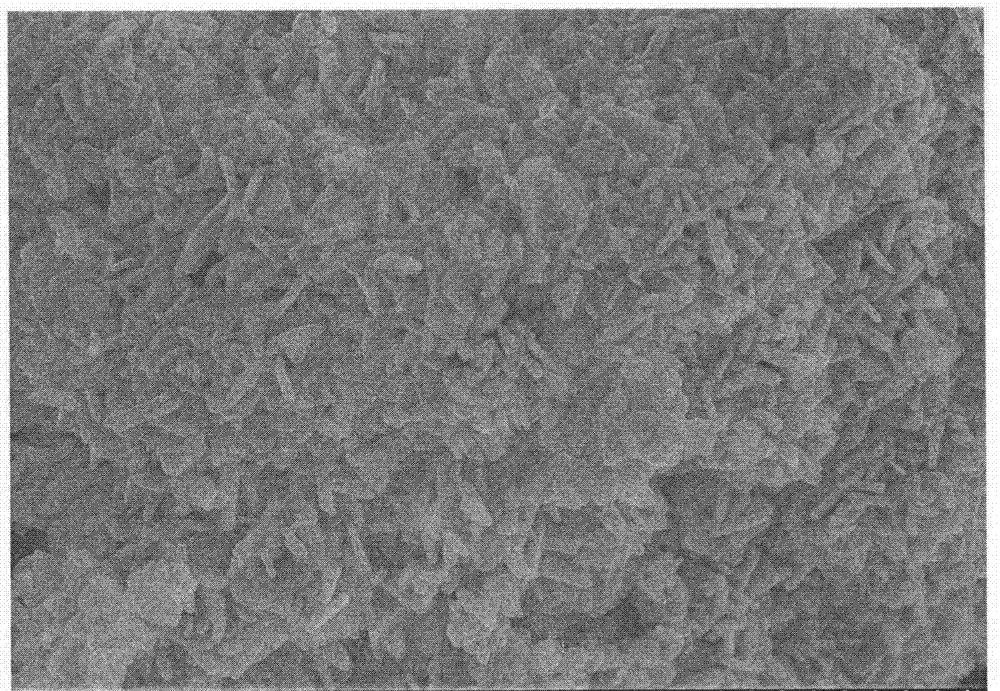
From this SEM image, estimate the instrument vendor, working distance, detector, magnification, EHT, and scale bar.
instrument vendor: Hitachi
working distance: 9 mm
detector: SE(M)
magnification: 20 K X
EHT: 10 kV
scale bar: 2000 nm